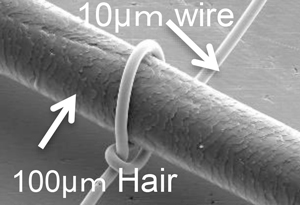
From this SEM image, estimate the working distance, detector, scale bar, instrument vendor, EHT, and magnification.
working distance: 20.7 mm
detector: SE(M)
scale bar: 100000 nm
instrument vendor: Hitachi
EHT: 15 kV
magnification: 0.5 K X